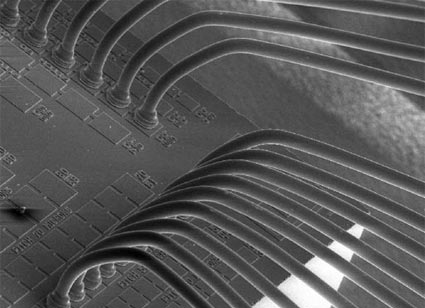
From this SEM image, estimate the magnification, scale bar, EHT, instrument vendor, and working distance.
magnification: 0.17 K X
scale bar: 200000 nm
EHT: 15 kV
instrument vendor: JEOL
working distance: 33 mm